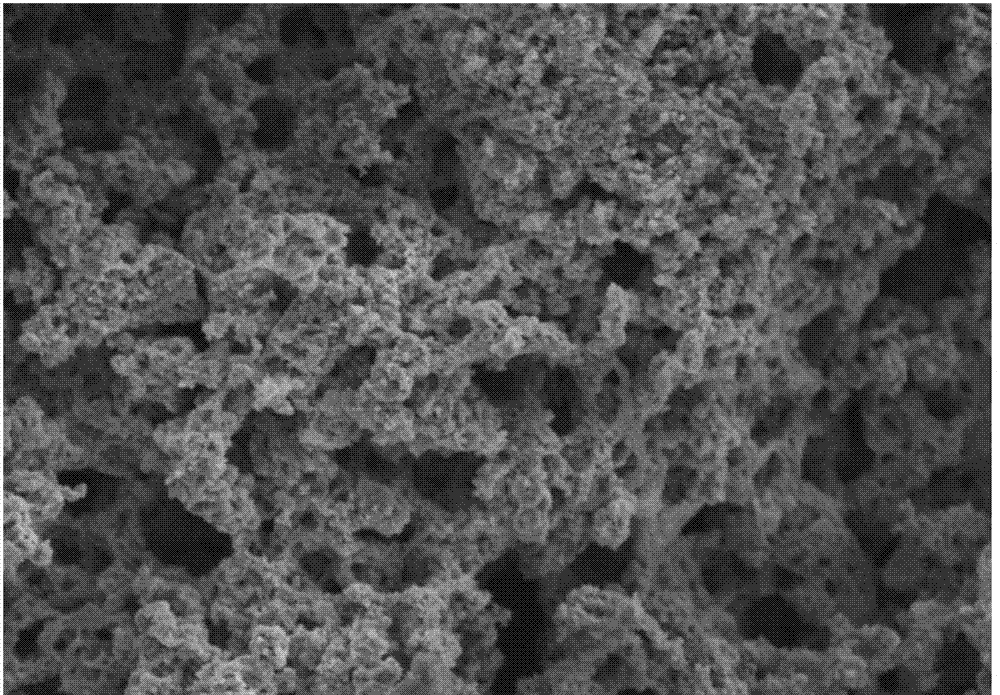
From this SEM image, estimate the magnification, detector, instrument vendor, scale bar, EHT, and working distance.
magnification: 5 K X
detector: SE(M)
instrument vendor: Hitachi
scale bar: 10000 nm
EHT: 5 kV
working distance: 9.9 mm